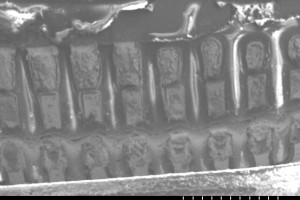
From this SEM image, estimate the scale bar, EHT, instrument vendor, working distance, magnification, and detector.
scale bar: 1000000 nm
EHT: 15 kV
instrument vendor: Hitachi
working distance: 55.5 mm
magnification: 0.04 K X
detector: SE